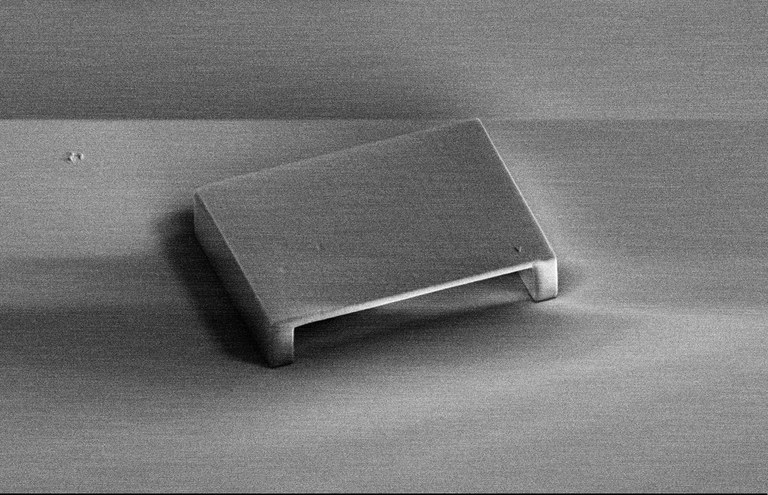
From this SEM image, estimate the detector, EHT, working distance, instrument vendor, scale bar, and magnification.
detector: SE2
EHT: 5 kV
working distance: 22.2 mm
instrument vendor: Zeiss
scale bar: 10000 nm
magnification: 0.768 K X